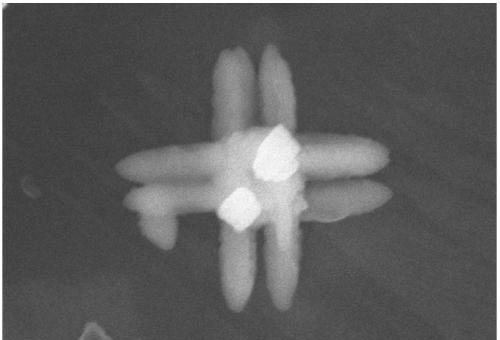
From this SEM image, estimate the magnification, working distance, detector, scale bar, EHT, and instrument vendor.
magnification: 80 K X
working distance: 8.9 mm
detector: SE(UL)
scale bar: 500 nm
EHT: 5 kV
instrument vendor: Hitachi